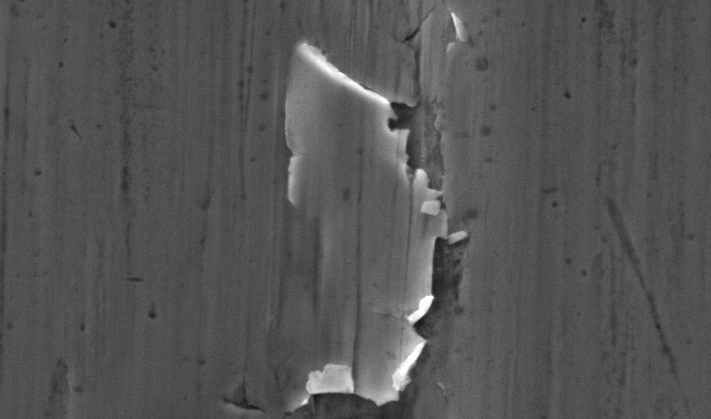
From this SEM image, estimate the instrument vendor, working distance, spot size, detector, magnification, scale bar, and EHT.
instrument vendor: FEI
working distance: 10.3 mm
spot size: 4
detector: SE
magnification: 2 K X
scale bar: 10000 nm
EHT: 20 kV